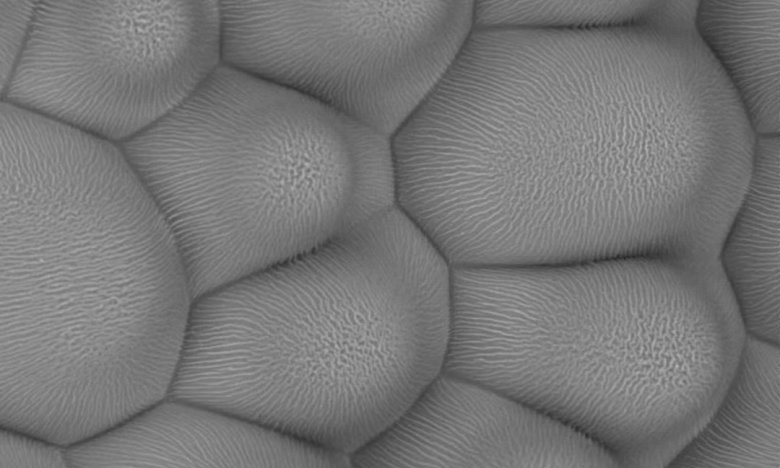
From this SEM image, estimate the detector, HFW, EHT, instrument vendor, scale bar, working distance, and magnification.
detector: BSD Full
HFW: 150 µm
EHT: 10 kV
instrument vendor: Thermo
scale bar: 30000 nm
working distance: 7.148 mm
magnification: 0.85 K X